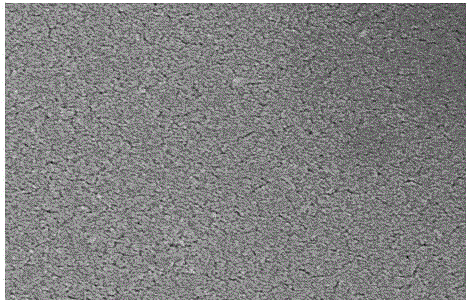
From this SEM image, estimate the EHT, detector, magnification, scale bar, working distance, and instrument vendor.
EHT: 3 kV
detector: SE(M)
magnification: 20 K X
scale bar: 2000 nm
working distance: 9.4 mm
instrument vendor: Hitachi